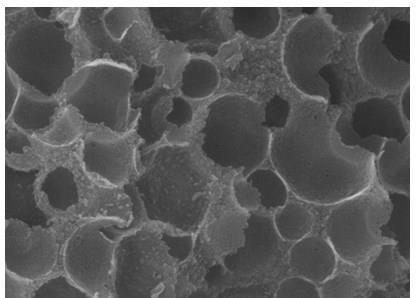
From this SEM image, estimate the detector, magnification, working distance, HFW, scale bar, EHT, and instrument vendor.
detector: In-Beam SE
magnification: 110 K X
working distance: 9.29 mm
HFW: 2.54 µm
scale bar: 200 nm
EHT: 20 kV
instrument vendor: Tescan